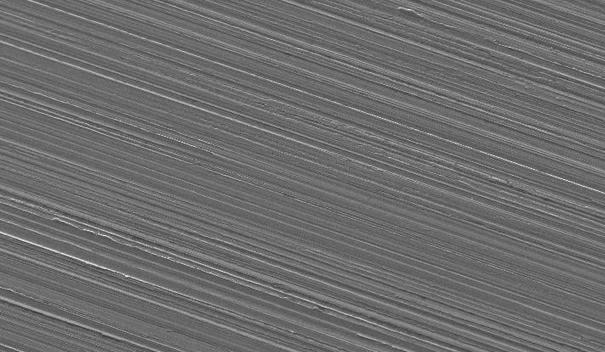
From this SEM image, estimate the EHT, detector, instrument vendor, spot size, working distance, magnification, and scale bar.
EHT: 20 kV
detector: SE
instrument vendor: FEI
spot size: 3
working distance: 9.9 mm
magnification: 0.163 K X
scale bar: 200000 nm